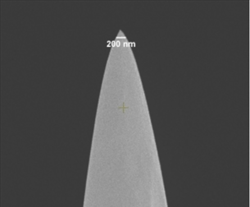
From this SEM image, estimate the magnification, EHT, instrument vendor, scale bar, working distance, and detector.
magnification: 60 K X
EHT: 3 kV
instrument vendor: FEI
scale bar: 2000 nm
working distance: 4.9 mm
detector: TLD